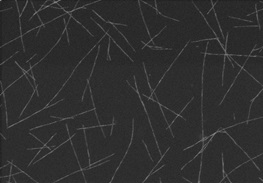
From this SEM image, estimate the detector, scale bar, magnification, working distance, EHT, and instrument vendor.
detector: SE(M,LA0)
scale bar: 3000 nm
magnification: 18 K X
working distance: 7.9 mm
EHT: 5 kV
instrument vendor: Hitachi